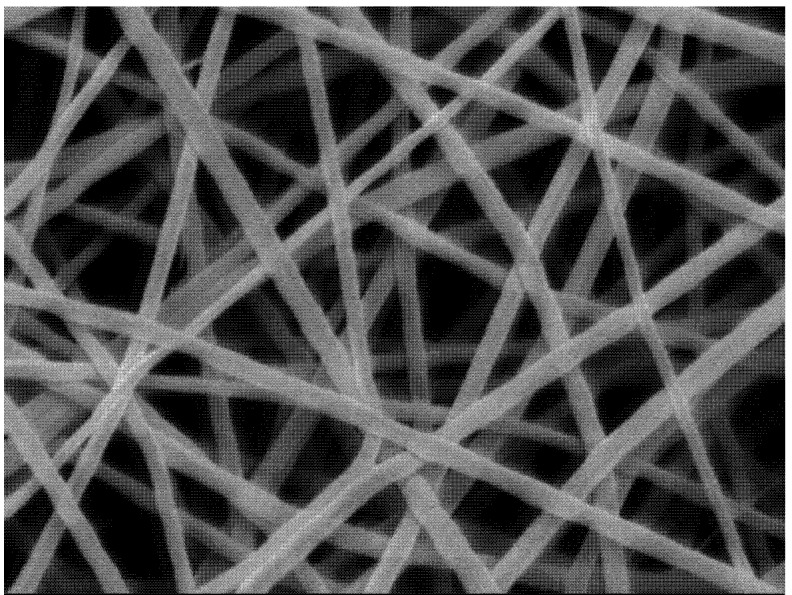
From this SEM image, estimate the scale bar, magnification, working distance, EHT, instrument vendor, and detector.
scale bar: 1000 nm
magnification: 20 K X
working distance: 9 mm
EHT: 5 kV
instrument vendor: JEOL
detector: SEI